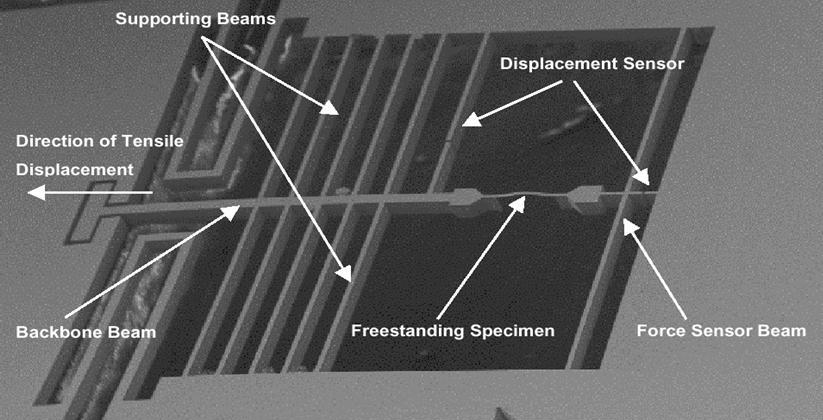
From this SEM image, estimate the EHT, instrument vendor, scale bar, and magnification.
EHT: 5 kV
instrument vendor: FEI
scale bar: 500000 nm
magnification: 0.085 K X